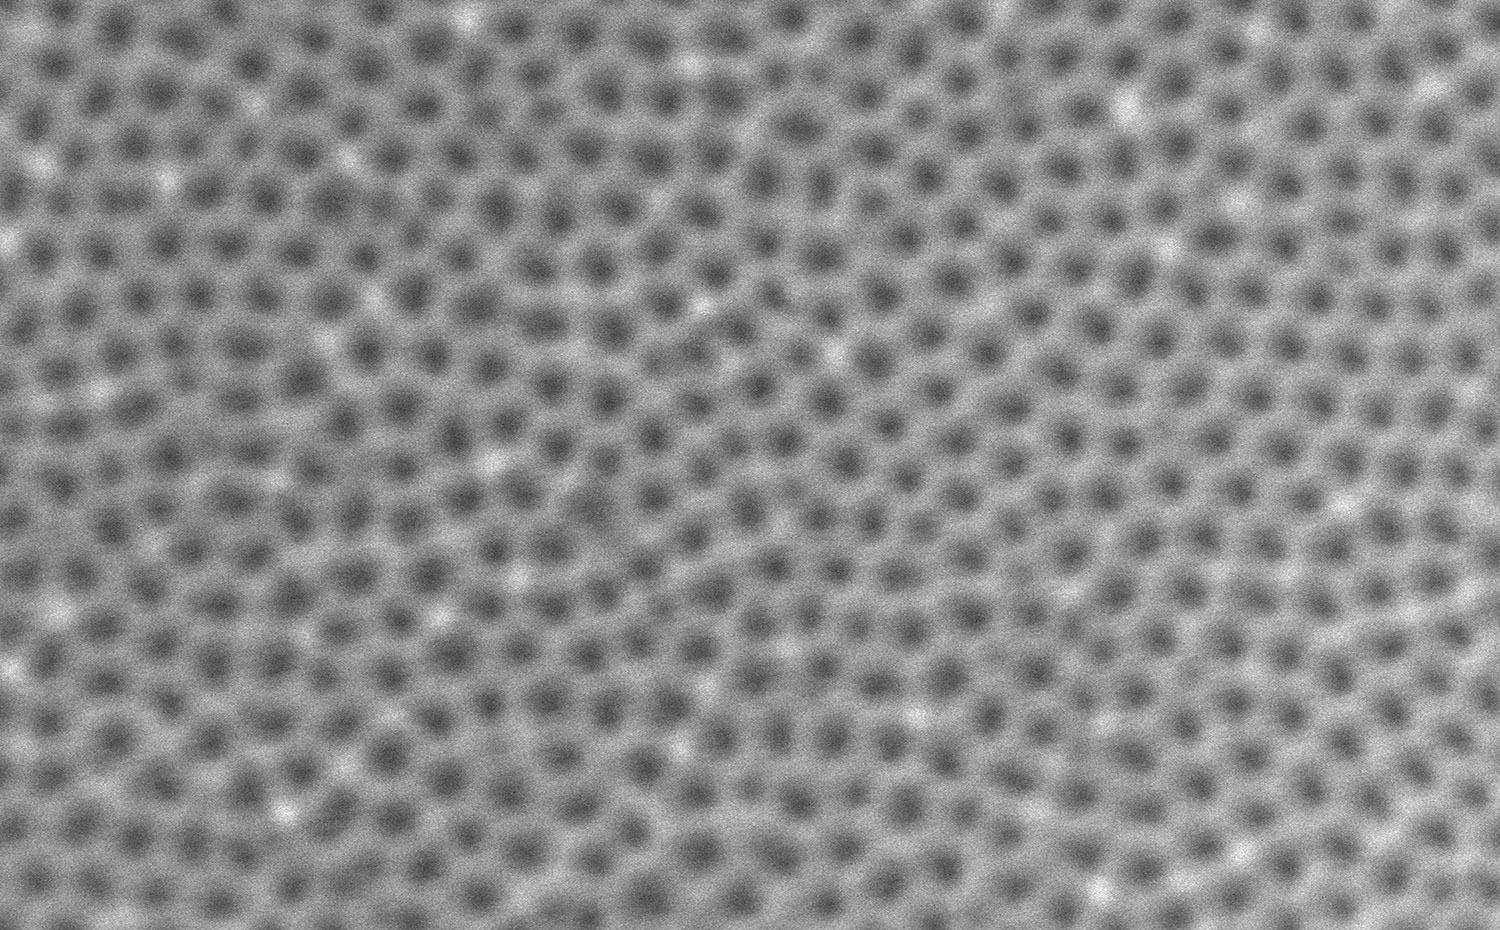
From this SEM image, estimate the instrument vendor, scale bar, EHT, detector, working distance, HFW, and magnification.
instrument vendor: other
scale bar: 800 nm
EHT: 10 kV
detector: SED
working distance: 6.504 mm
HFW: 2.59 µm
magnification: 200 K X